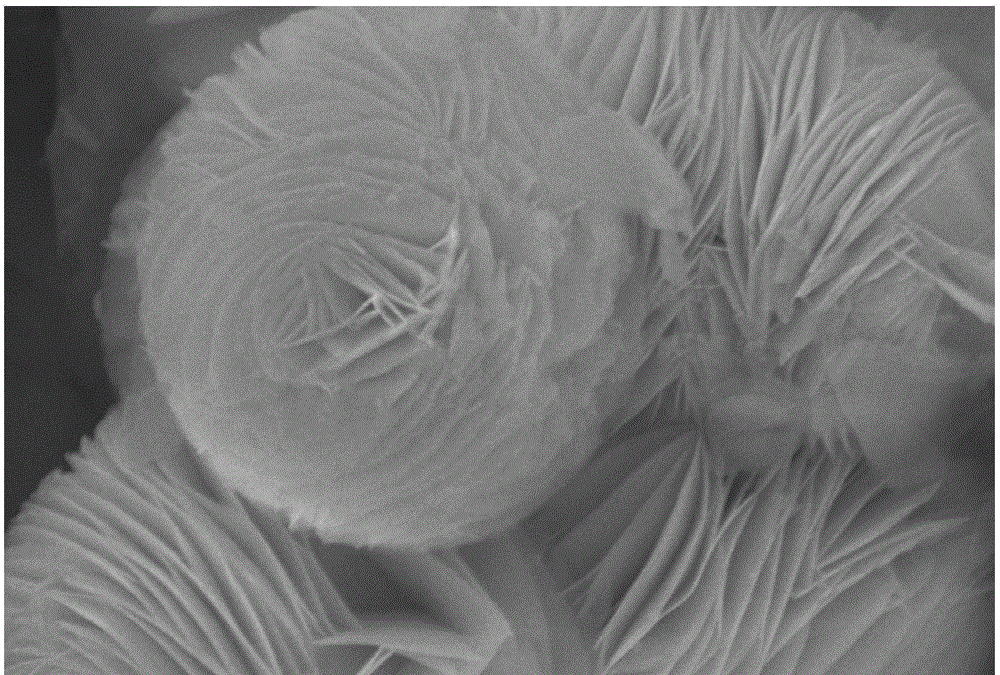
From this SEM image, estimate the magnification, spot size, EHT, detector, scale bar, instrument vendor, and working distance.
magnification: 10 K X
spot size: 35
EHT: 30 kV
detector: SEI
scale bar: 1000 nm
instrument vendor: JEOL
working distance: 10 mm